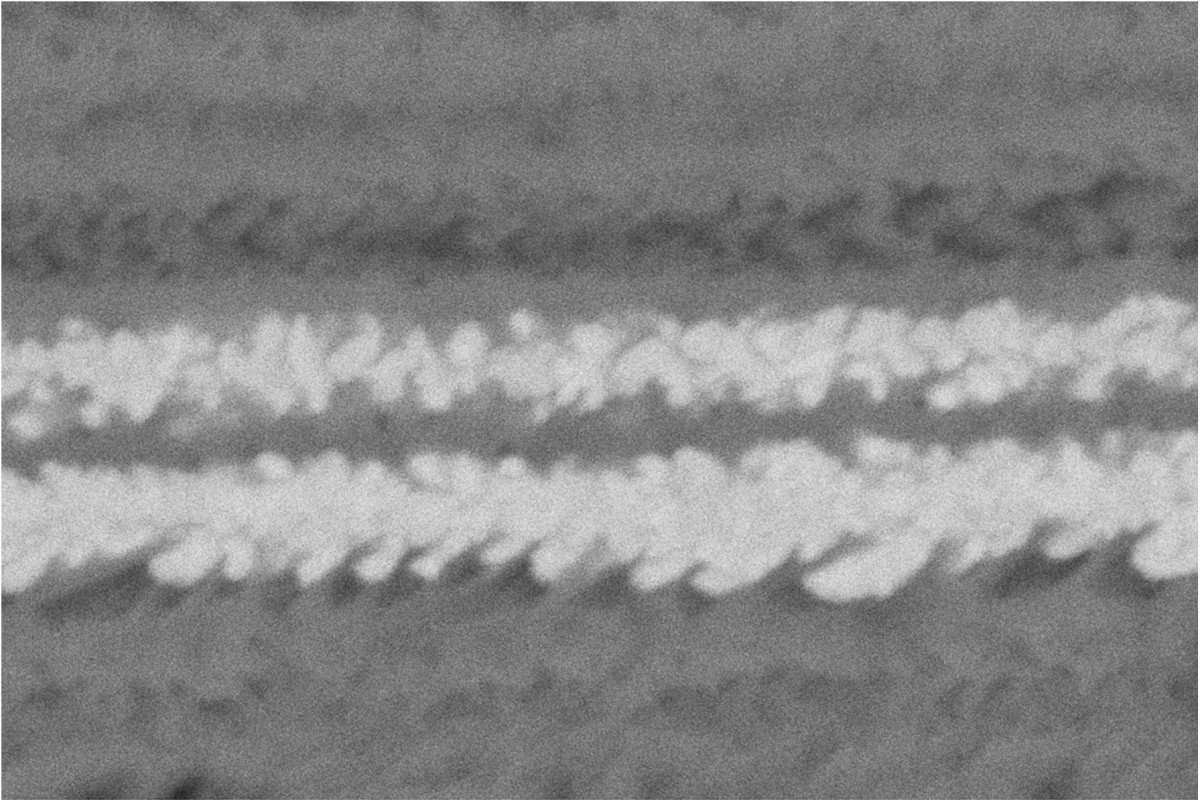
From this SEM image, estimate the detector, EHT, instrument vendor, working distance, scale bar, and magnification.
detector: ESB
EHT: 3 kV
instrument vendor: Zeiss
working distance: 4 mm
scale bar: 100 nm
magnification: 100 K X